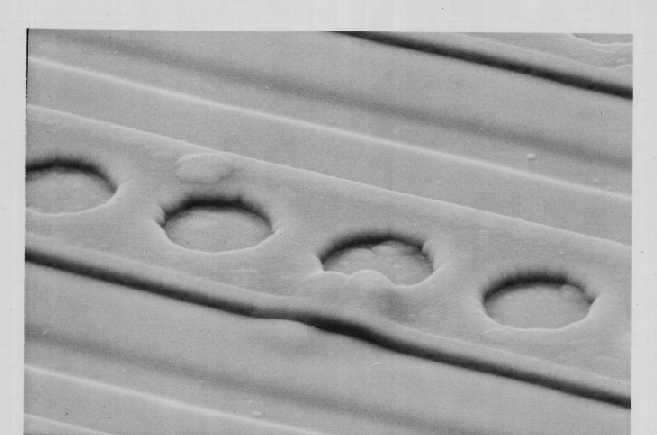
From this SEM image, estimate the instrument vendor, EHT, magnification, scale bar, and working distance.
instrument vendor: JEOL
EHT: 15 kV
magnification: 3.5 K X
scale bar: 10000 nm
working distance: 39 mm